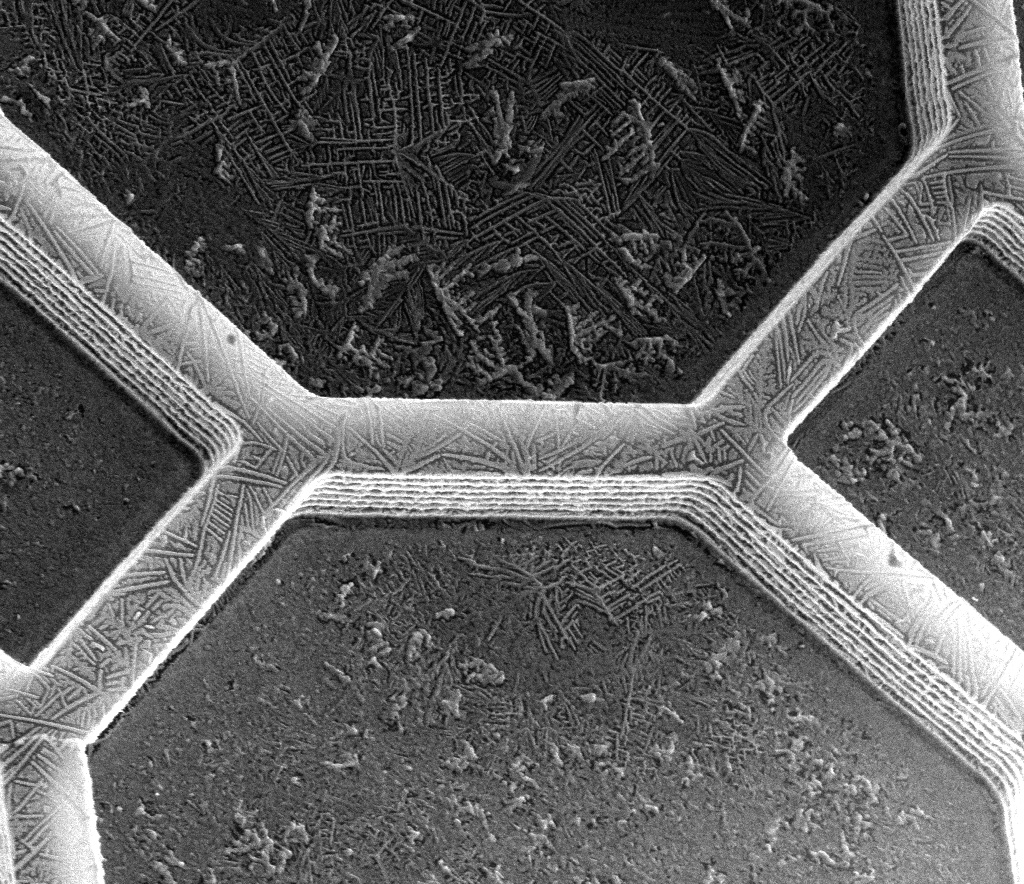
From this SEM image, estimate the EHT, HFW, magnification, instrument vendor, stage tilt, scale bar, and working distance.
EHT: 5 kV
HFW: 51.2 µm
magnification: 2.5 K X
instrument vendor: FEI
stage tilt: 0°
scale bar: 10000 nm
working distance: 9.1 mm